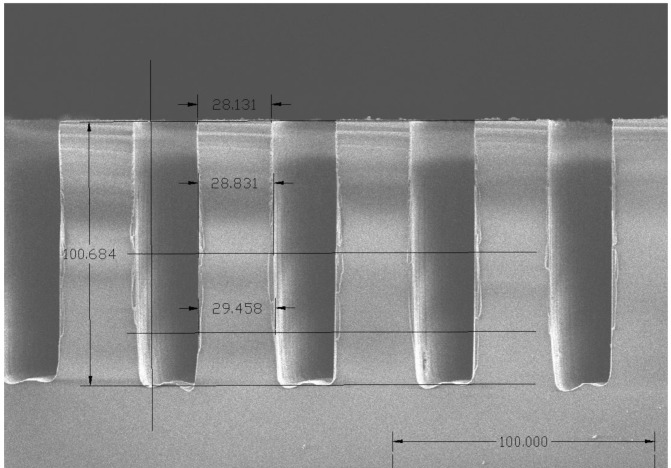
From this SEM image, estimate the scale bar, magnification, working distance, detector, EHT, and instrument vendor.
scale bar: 100000 nm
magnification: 0.5 K X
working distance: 12.4 mm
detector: SE(M)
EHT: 5 kV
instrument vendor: Hitachi